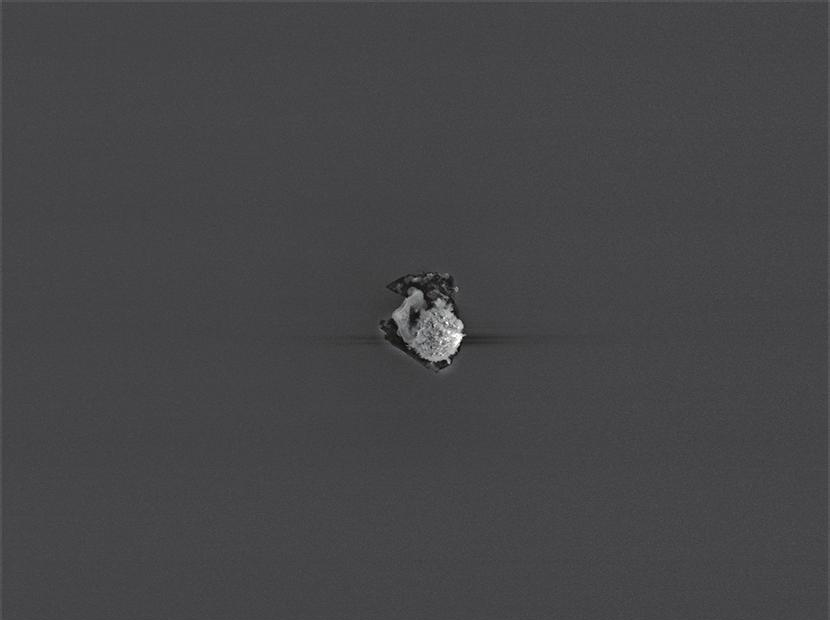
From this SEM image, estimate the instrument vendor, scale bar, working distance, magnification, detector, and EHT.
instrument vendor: JEOL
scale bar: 10000 nm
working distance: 14.9 mm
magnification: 0.85 K X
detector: SEI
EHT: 15 kV